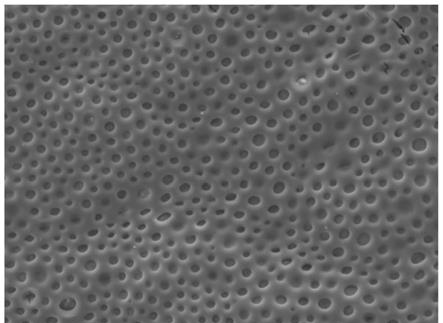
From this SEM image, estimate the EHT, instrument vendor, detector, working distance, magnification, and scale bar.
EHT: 10 kV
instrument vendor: JEOL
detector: LED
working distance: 7.8 mm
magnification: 1 K X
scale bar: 10000 nm